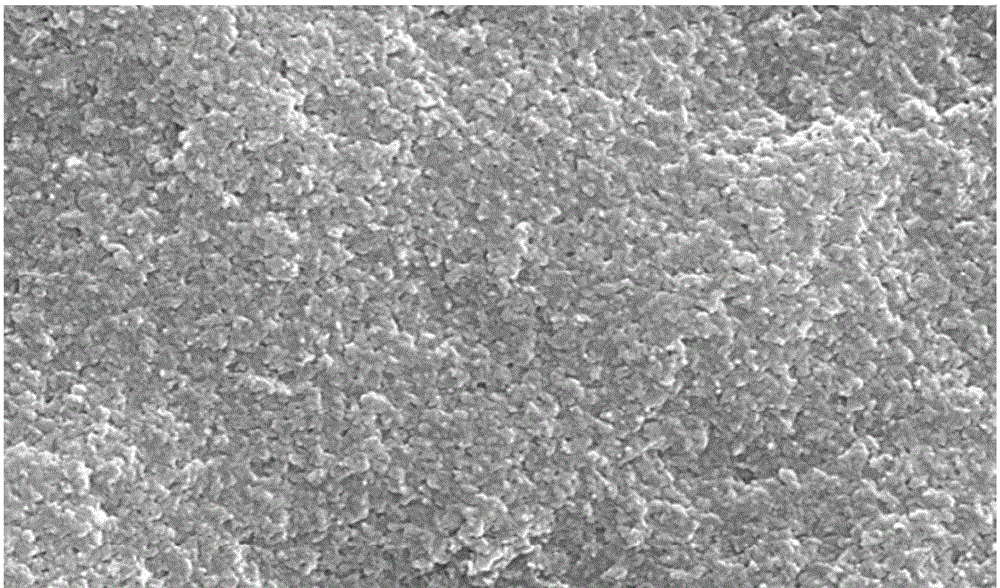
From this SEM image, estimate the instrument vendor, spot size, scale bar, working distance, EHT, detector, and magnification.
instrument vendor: FEI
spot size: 4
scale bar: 2000 nm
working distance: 7.3 mm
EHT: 25 kV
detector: SE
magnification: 20 K X